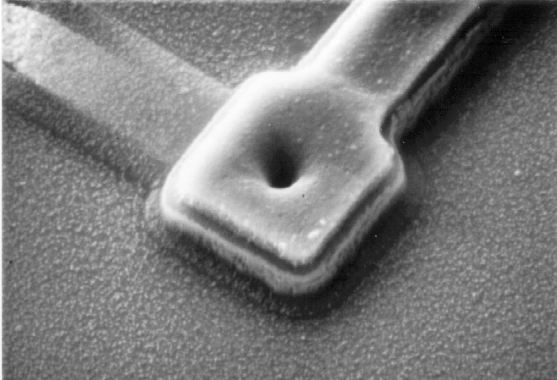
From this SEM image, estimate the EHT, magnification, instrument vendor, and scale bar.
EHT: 10 kV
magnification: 10 K X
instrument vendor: other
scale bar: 1000 nm